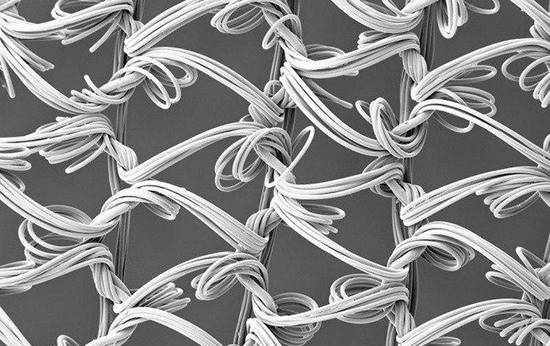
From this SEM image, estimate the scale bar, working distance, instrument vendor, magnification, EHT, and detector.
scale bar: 100000 nm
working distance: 19.4 mm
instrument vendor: Zeiss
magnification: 0.05 K X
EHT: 5 kV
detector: SE2